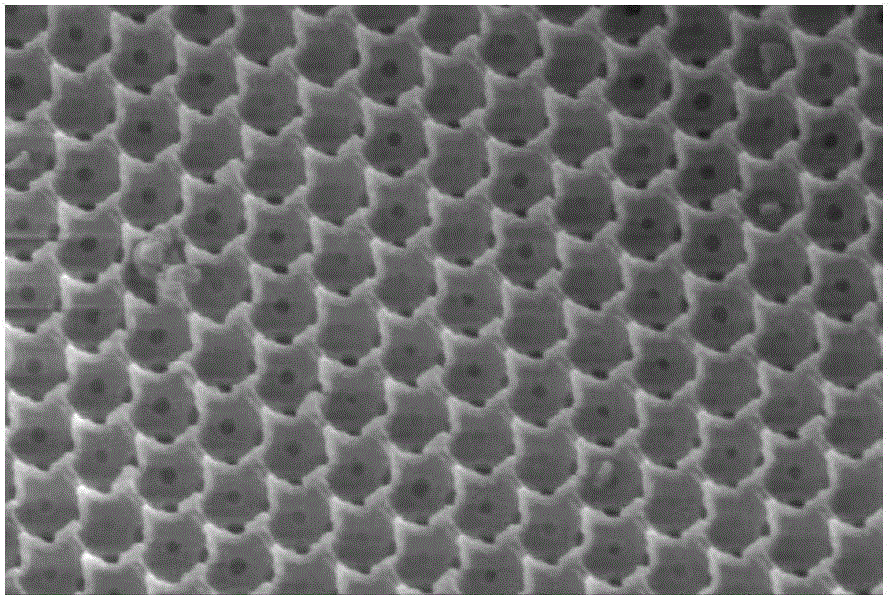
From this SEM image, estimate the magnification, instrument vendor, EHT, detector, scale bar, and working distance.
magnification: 50 K X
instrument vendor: Hitachi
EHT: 20 kV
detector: SE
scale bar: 1000 nm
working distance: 14.6 mm